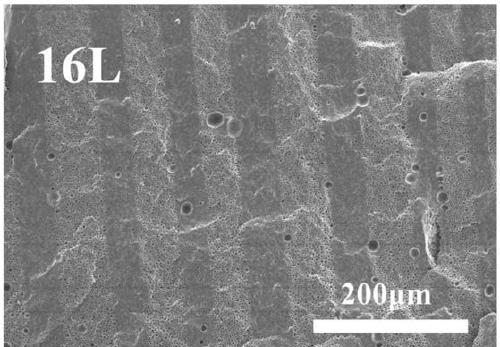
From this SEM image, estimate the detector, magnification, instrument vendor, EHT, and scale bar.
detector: SED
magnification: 0.2 K X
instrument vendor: other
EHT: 10 kV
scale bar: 200000 nm